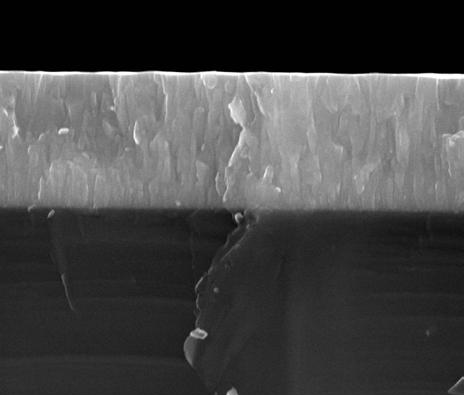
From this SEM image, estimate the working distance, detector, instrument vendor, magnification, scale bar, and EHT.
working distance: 10 mm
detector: vCD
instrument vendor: FEI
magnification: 100 K X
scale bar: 1000 nm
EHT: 15 kV